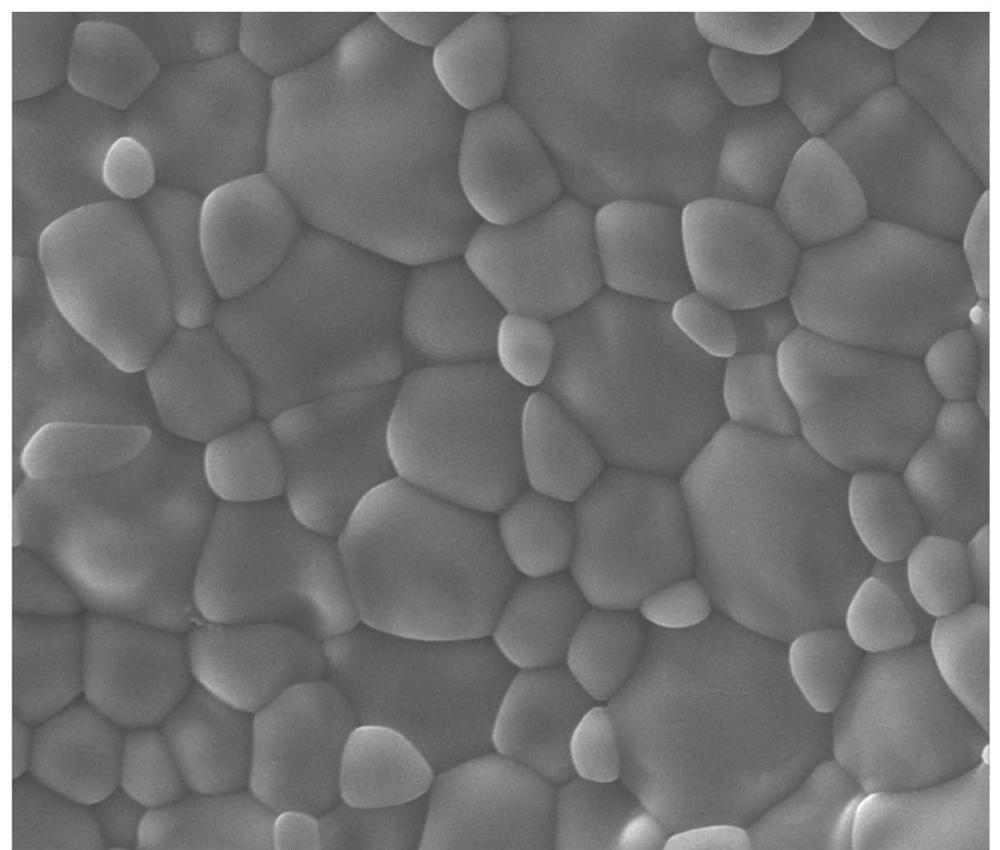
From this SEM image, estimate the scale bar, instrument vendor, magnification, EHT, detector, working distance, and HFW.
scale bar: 10000 nm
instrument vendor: FEI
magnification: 2.5 K X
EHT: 20 kV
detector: ETD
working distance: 12.1 mm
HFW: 54.08 µm